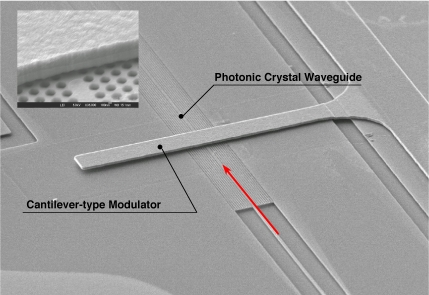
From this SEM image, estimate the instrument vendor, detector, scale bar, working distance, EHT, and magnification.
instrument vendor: JEOL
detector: LEI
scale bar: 10000 nm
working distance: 15.1 mm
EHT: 5 kV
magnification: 1.4 K X